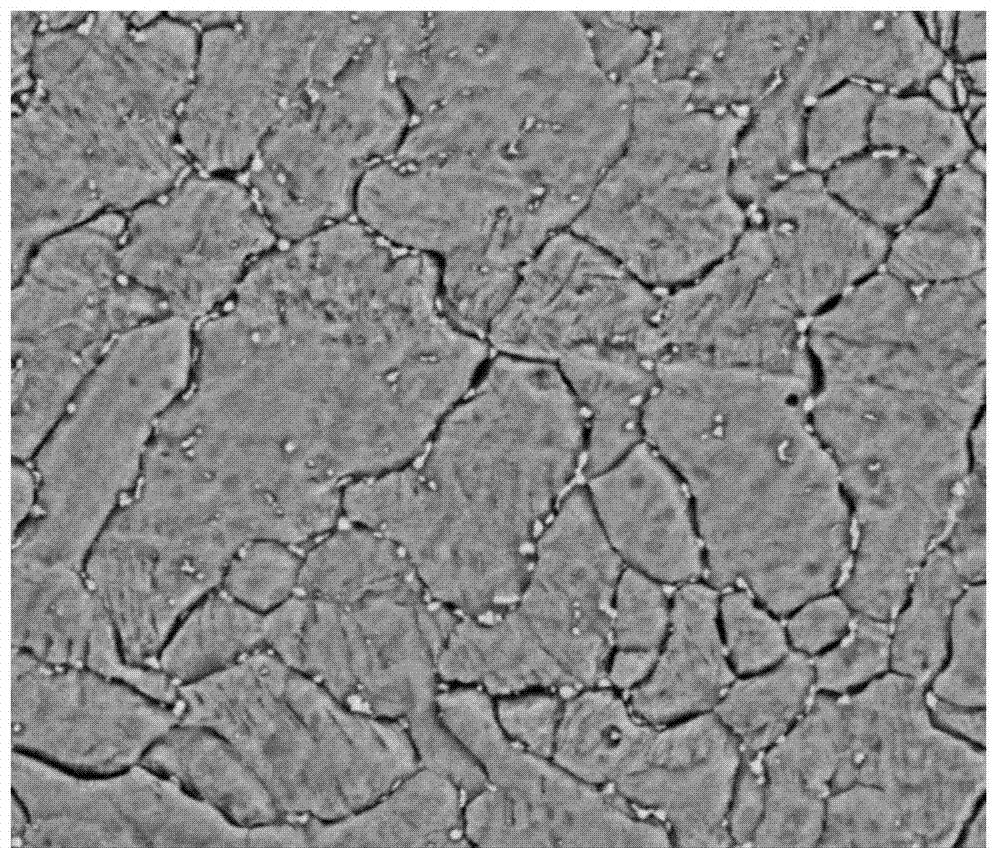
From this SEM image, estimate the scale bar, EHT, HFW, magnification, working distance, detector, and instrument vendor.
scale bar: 10000 nm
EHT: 20 kV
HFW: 48 µm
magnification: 5 K X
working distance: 6.2 mm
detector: BSED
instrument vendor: FEI